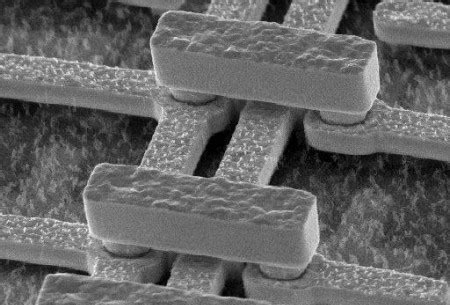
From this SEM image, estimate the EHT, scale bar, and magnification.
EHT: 20 kV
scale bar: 8760 nm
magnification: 3.5 K X